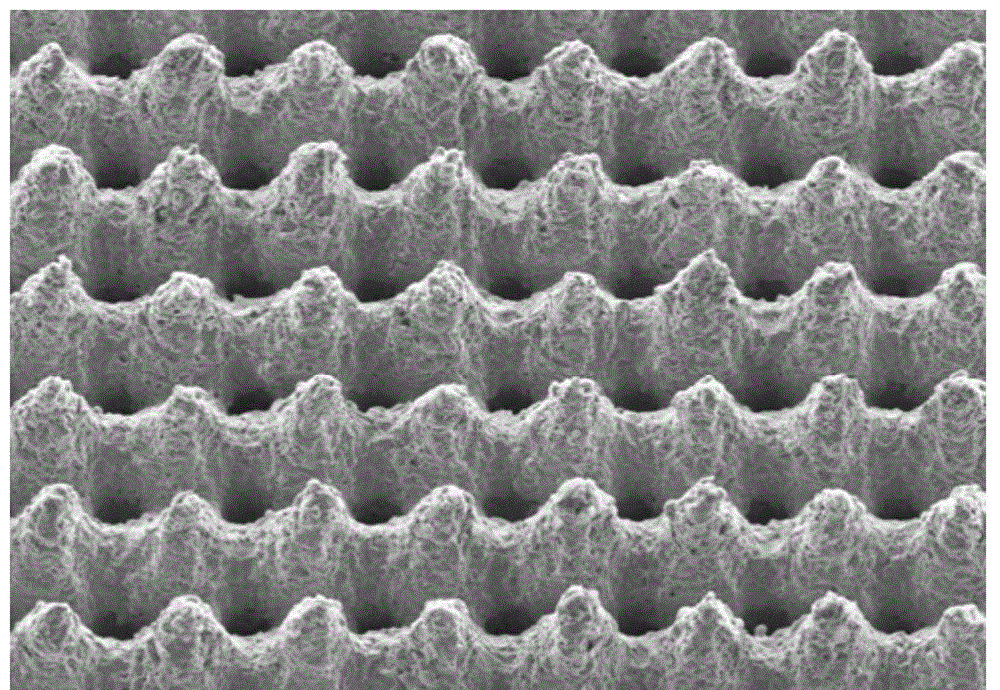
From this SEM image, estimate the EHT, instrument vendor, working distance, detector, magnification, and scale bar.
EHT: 20 kV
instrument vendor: Hitachi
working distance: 14.9 mm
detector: SE(M)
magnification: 0.2 K X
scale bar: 200000 nm